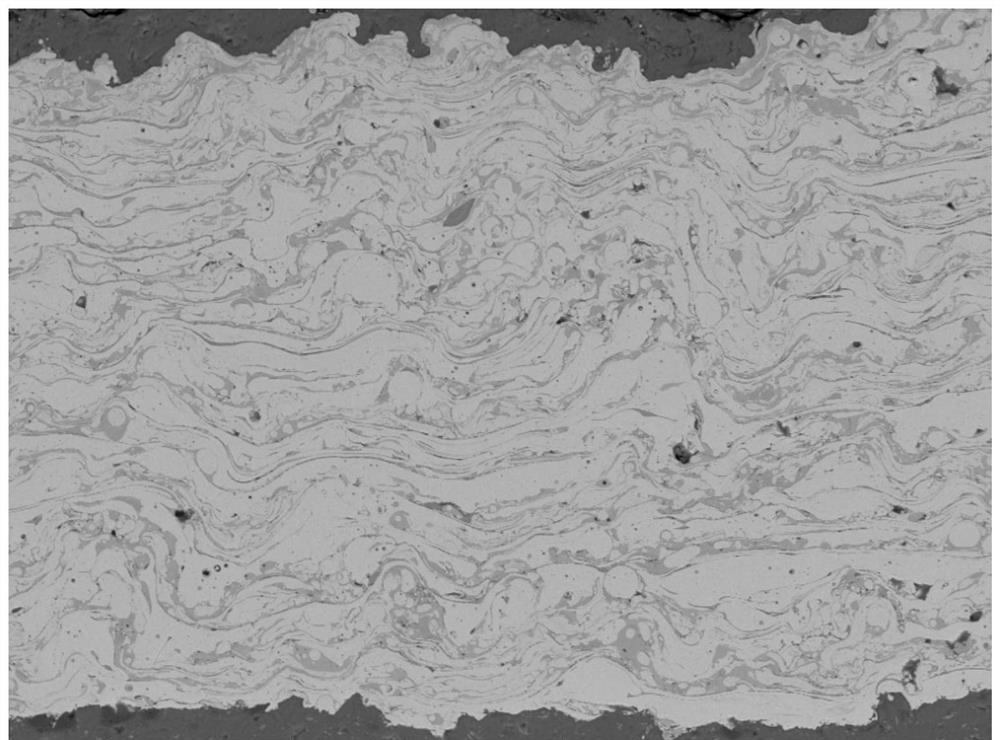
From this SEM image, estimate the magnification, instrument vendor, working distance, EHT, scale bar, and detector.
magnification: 0.5 K X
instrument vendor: FEI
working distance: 15.6 mm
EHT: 20 kV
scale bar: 200000 nm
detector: DualBSD